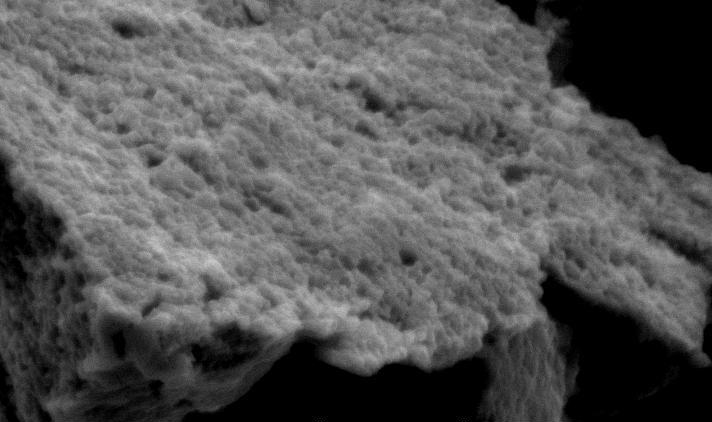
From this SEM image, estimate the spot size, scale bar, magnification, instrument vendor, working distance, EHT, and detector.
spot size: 4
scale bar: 5000 nm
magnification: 5 K X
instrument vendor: FEI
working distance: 10 mm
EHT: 20 kV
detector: SE